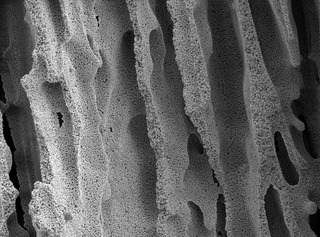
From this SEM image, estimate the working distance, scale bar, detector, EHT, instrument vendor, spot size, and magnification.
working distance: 7.2 mm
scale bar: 10000 nm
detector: SE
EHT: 5 kV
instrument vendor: FEI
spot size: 3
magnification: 2.147 K X